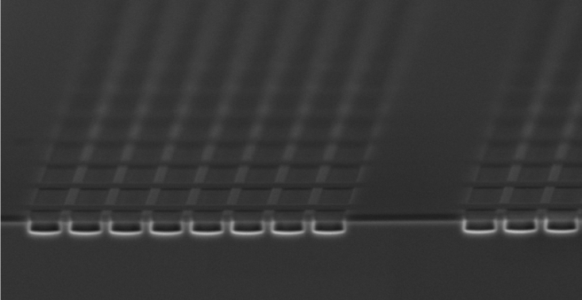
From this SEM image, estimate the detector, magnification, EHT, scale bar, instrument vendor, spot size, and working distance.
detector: SEI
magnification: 3.3 K X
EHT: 10 kV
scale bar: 5000 nm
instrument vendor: JEOL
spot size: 48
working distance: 21 mm